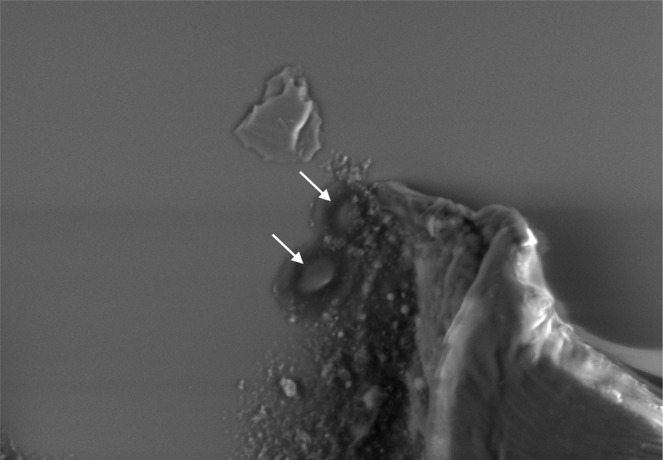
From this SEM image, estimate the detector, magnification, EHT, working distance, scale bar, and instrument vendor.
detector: BSE M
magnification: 2.5 K X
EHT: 10 kV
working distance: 5.2 mm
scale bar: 20000 nm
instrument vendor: Hitachi